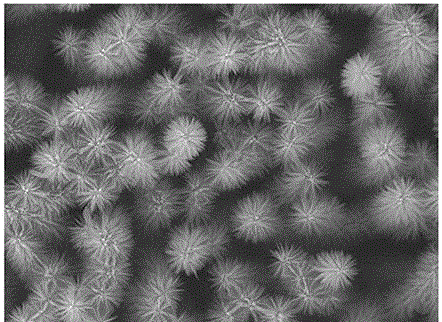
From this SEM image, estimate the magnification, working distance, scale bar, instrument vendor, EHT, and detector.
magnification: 3 K X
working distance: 8 mm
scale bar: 1000 nm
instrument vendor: JEOL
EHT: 15 kV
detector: SEI